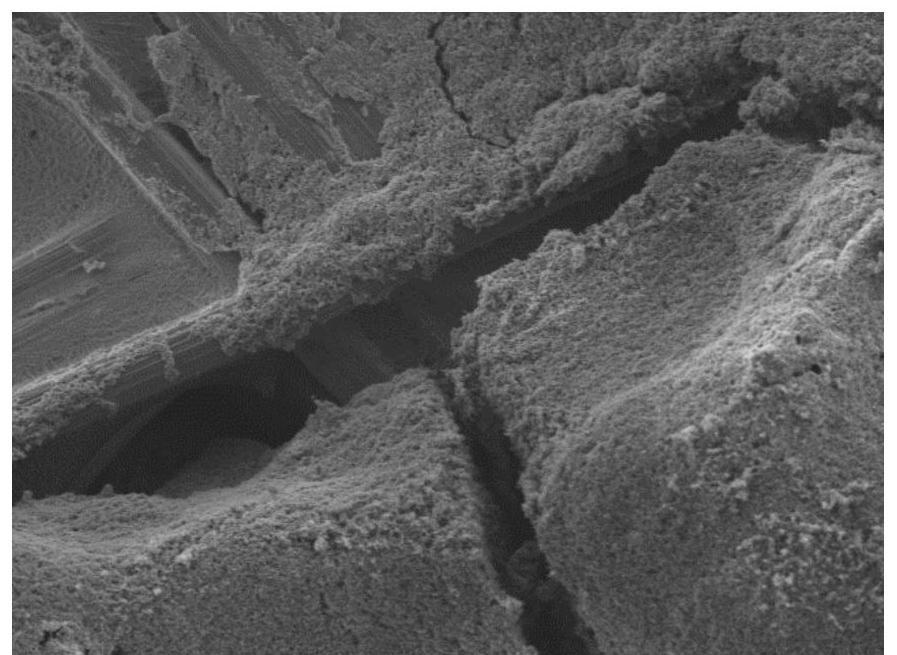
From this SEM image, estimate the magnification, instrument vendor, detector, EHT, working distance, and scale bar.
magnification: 1 K X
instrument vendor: JEOL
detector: LED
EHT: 3 kV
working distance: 8 mm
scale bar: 10000 nm